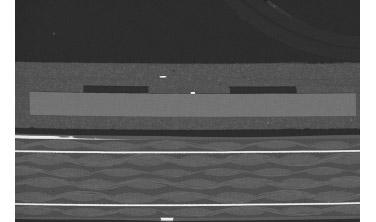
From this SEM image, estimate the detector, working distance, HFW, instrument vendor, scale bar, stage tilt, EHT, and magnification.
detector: T1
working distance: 51.4 mm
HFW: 5180 µm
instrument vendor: FEI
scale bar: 2000000 nm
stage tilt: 0°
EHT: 30 kV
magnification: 0.08 K X